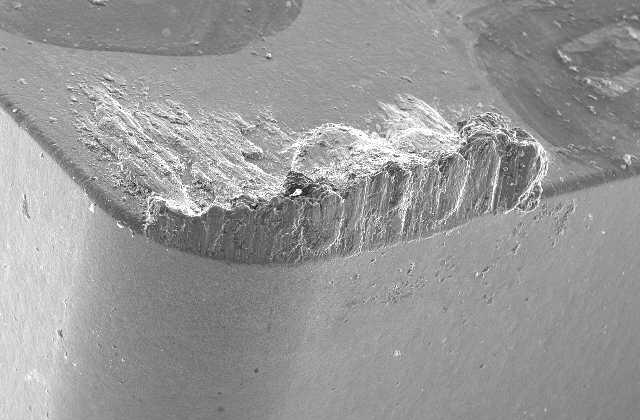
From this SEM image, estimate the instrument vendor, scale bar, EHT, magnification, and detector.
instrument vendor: JEOL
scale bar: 500000 nm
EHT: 20 kV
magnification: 0.04 K X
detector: SEI